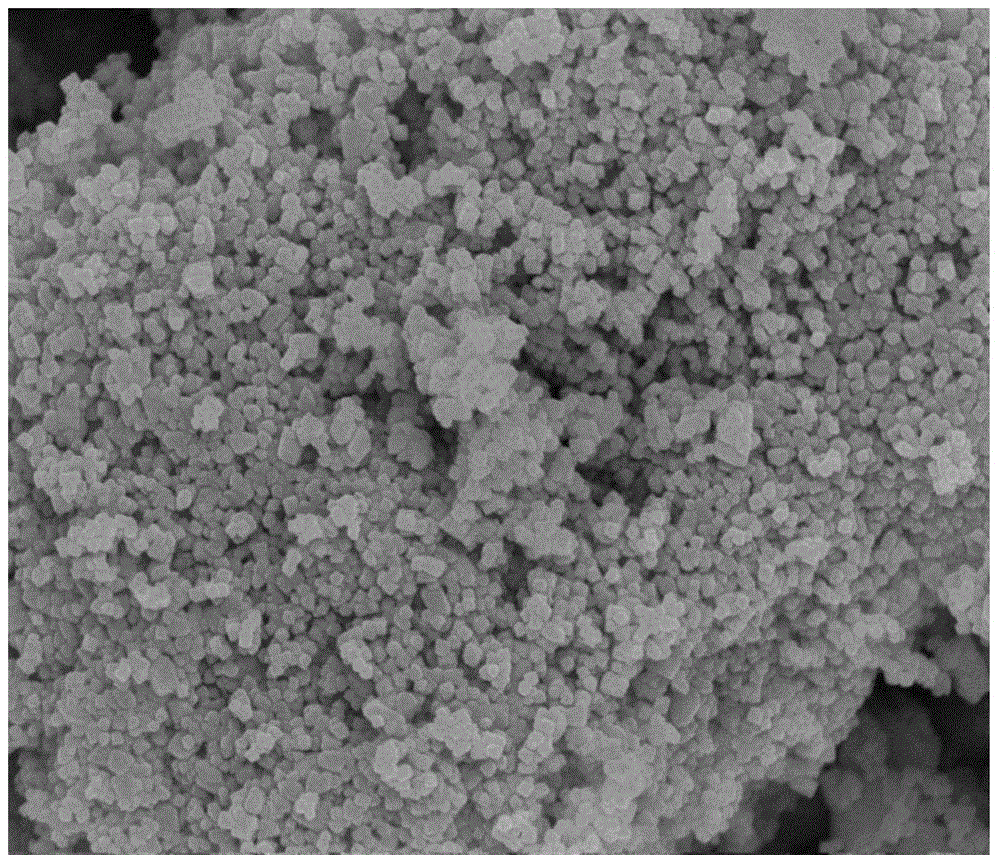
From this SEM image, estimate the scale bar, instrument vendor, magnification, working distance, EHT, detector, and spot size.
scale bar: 1000 nm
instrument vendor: FEI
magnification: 50 K X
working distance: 5.2 mm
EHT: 10 kV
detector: TLD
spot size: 3